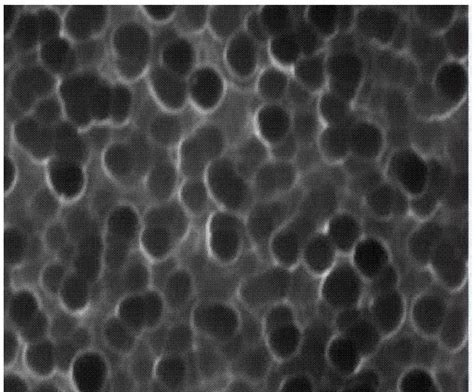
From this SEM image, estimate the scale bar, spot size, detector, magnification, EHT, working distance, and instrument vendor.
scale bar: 1000 nm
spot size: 40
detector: SEI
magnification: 15 K X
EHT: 25 kV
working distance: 10 mm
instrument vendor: JEOL